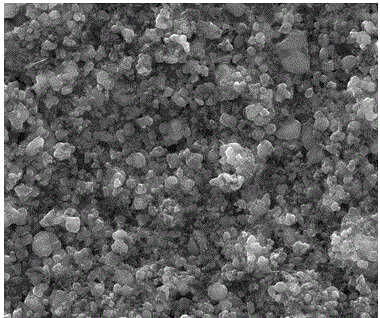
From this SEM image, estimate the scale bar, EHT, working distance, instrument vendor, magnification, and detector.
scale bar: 10000 nm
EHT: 25 kV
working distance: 12.1 mm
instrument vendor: FEI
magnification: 12 K X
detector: ETD